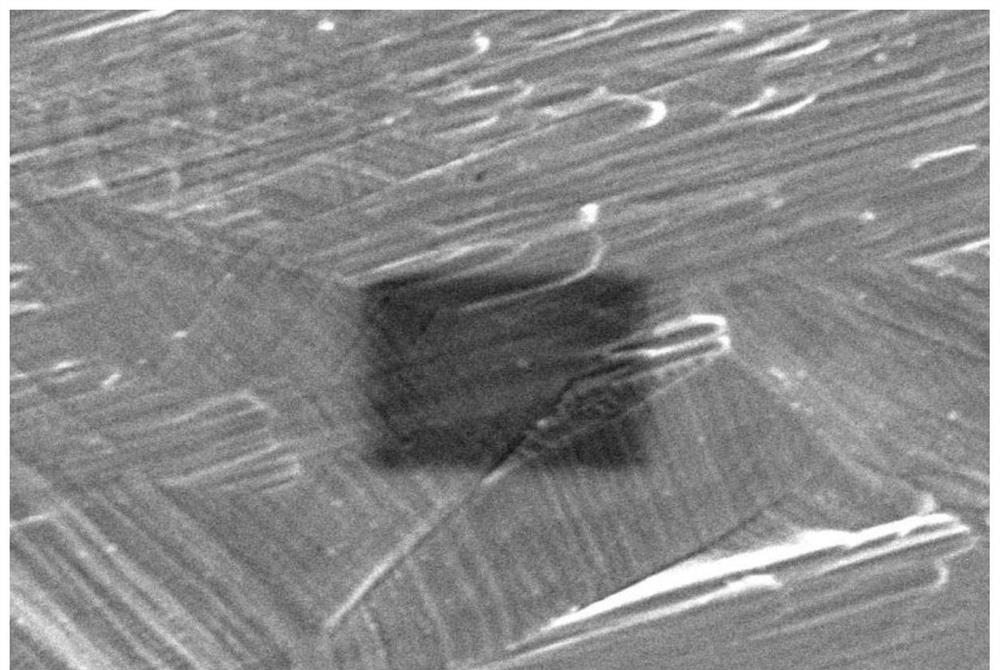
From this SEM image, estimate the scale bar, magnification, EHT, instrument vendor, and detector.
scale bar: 5000 nm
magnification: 5 K X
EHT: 10 kV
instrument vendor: JEOL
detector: SEI